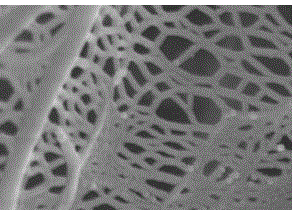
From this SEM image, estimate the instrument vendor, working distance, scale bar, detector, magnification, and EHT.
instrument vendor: JEOL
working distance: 4.2 mm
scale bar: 100 nm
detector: SEI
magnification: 100 K X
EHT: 5 kV